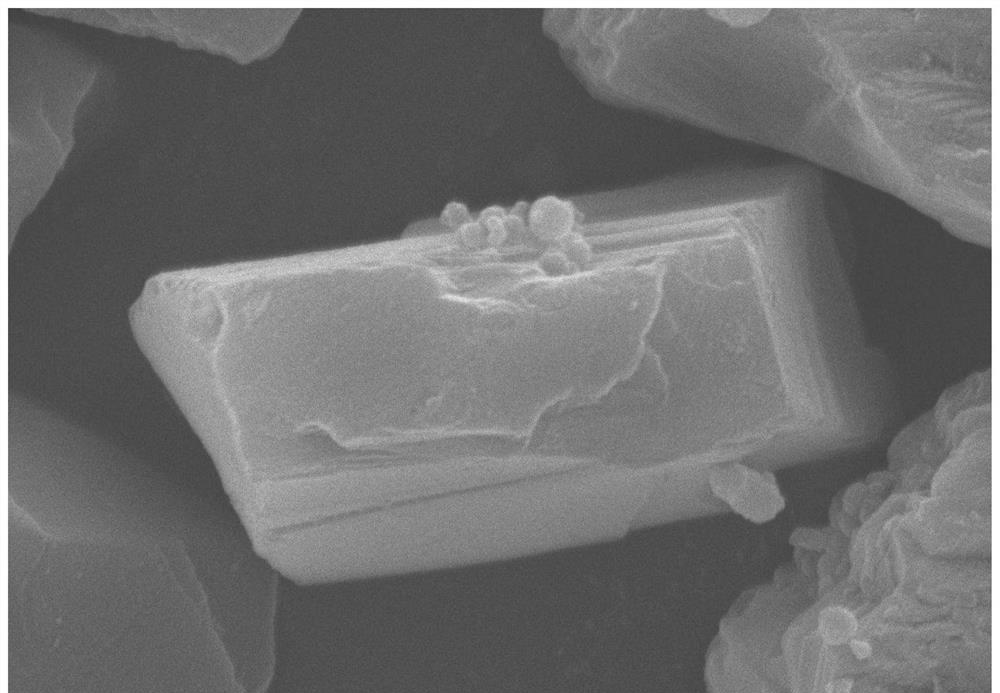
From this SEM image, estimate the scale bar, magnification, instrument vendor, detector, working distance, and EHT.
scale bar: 5000 nm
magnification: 10 K X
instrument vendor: Hitachi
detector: SE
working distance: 13.3 mm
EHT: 20 kV